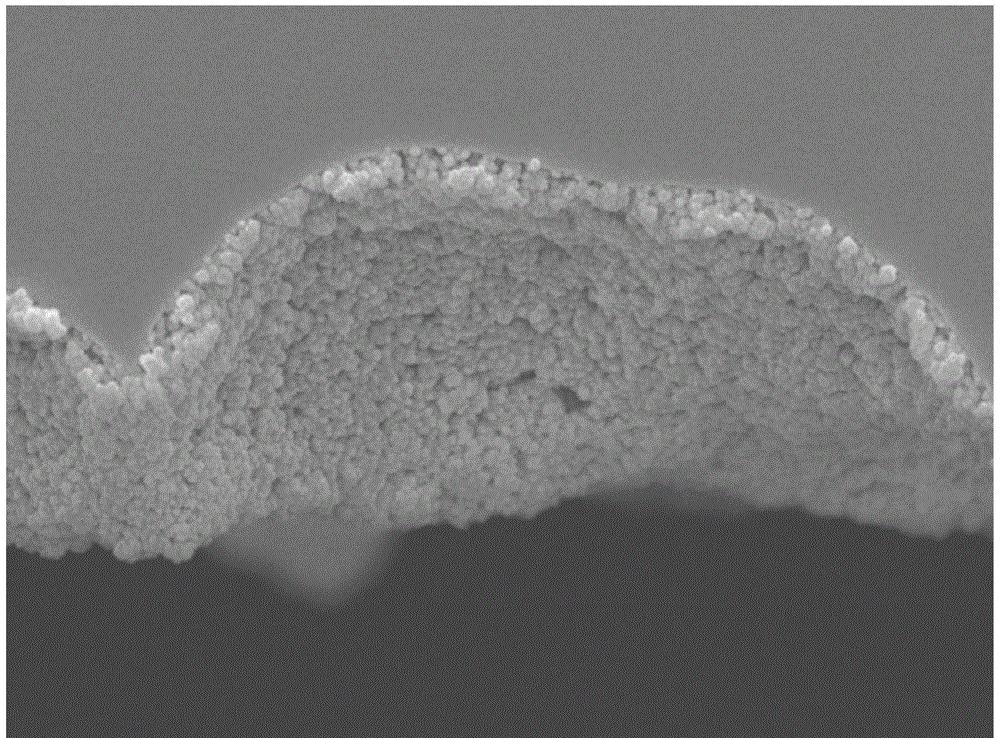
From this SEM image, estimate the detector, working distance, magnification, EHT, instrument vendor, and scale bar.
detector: SEI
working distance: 8.4 mm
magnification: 33 K X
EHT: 5 kV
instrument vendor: JEOL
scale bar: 100 nm